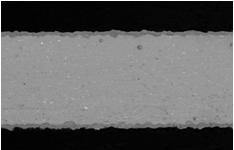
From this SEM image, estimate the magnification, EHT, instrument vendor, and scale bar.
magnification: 0.25 K X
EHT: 15 kV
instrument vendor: JEOL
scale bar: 100000 nm